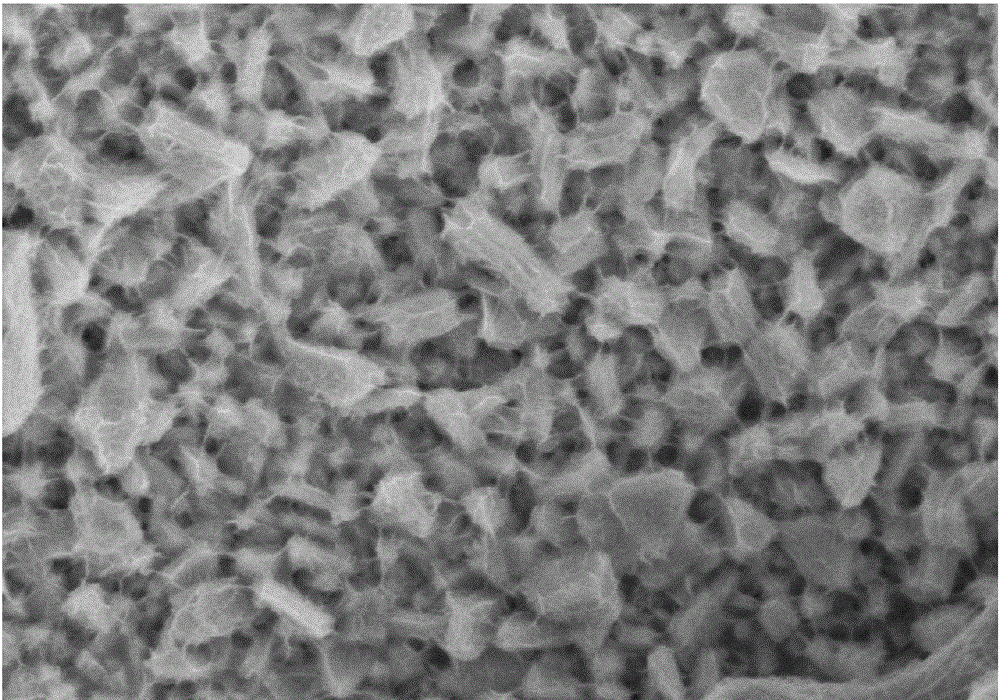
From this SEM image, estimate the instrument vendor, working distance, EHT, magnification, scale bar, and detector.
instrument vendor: Hitachi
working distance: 13.5 mm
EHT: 5 kV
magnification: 25 K X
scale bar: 2000 nm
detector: SE(M)